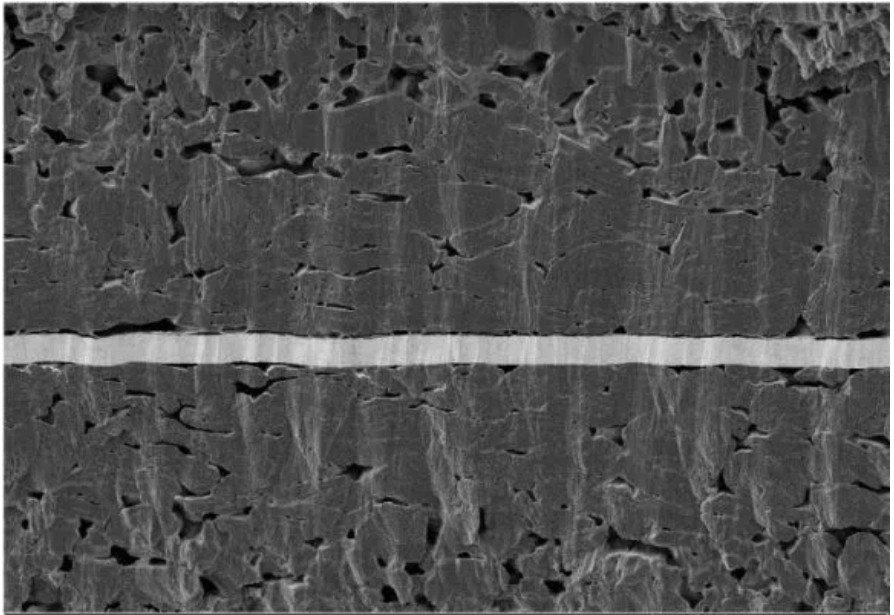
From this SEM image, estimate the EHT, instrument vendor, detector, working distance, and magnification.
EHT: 3 kV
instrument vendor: Zeiss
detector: SE2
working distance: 4.1 mm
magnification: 0.601 K X